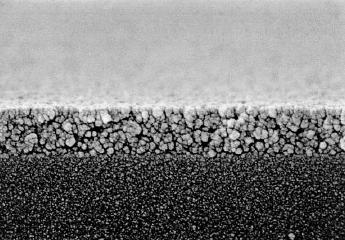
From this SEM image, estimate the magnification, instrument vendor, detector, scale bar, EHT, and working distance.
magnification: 100 K X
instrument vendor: Hitachi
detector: SE(U)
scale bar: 500 nm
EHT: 5 kV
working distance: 4 mm